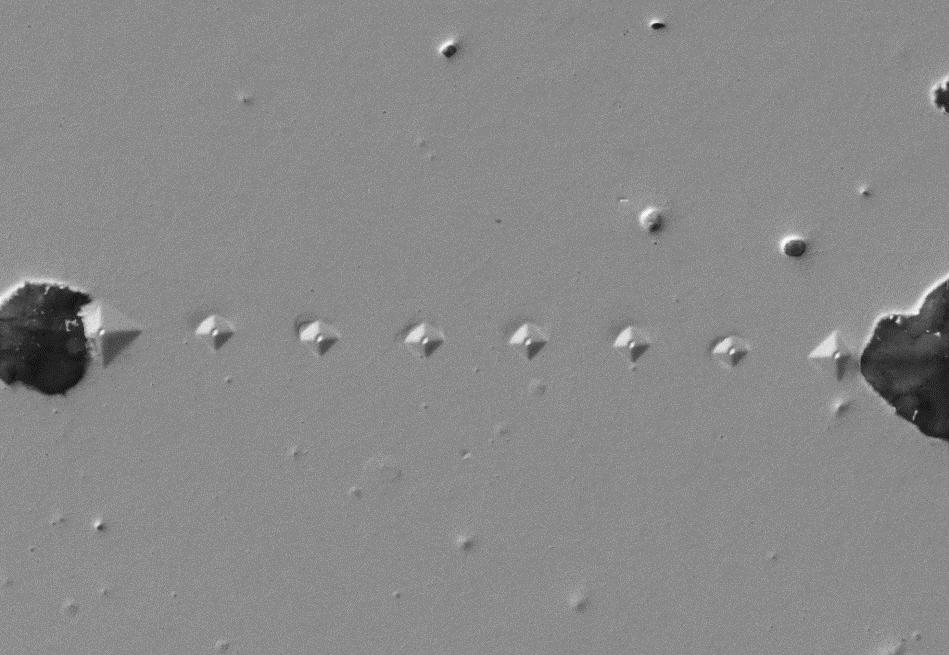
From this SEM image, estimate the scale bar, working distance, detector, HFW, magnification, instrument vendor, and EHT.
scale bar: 10000 nm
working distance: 10.1 mm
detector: SE2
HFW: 190.8 µm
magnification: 0.6 K X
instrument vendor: Zeiss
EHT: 20 kV